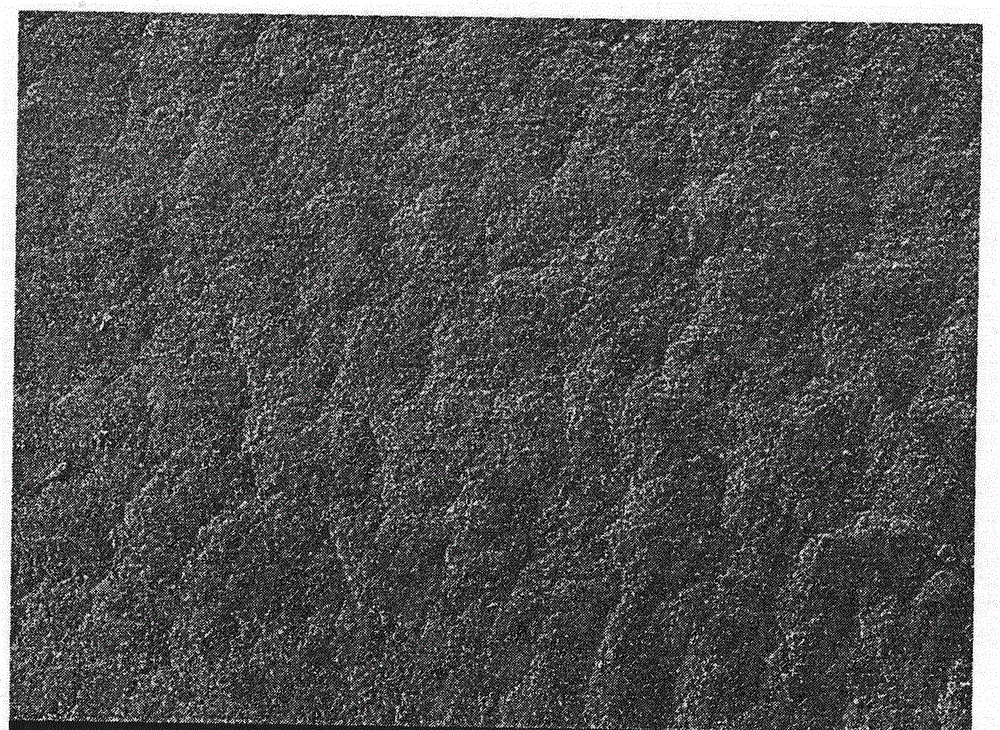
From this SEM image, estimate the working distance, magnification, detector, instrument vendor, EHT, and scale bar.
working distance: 8 mm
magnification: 0.1 K X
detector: SEI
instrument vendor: JEOL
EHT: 3 kV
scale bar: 100000 nm